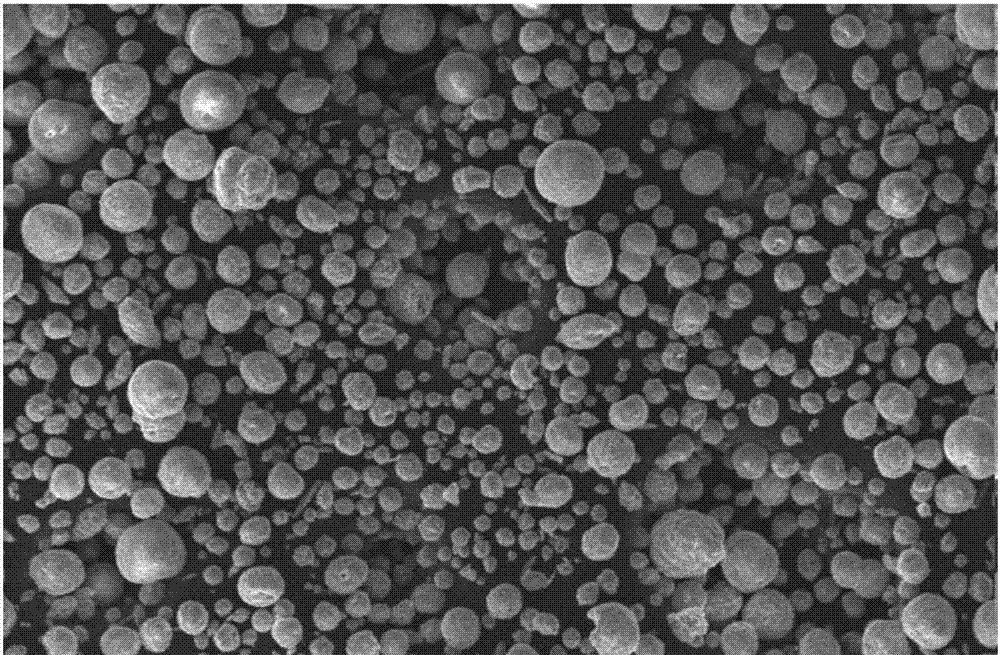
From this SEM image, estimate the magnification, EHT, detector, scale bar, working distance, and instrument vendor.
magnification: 0.1 K X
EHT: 10 kV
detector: SE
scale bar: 200000 nm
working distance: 11 mm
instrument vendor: FEI